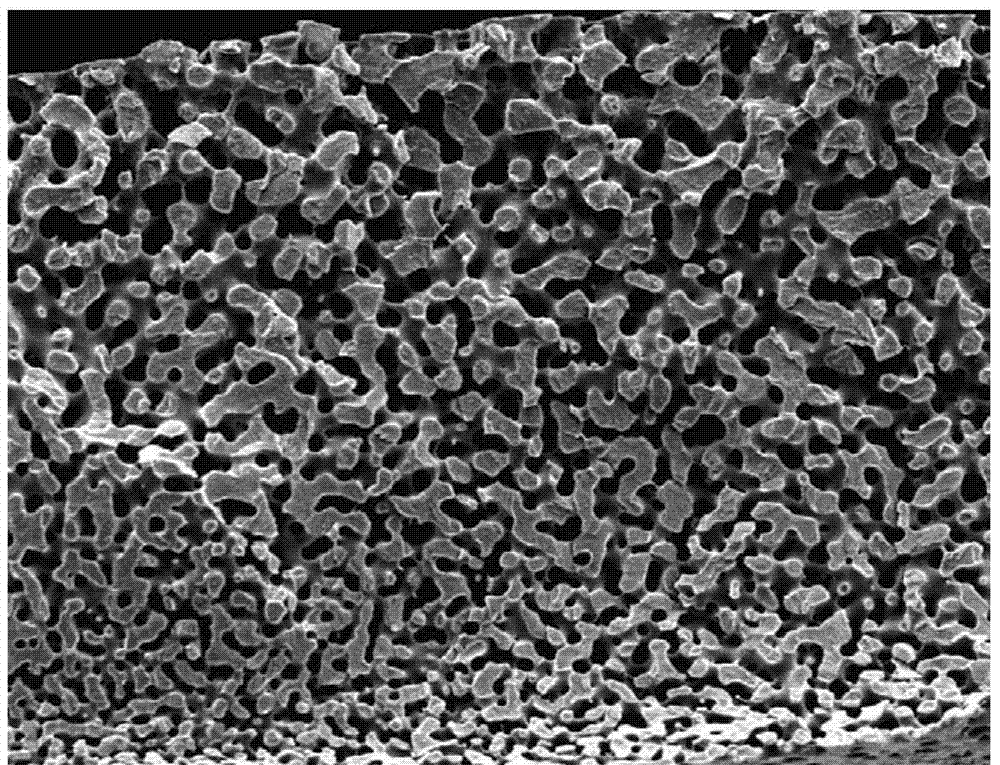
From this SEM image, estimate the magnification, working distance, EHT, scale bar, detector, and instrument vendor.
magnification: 0.2 K X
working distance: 10.9 mm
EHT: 20 kV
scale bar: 500000 nm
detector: SE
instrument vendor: FEI